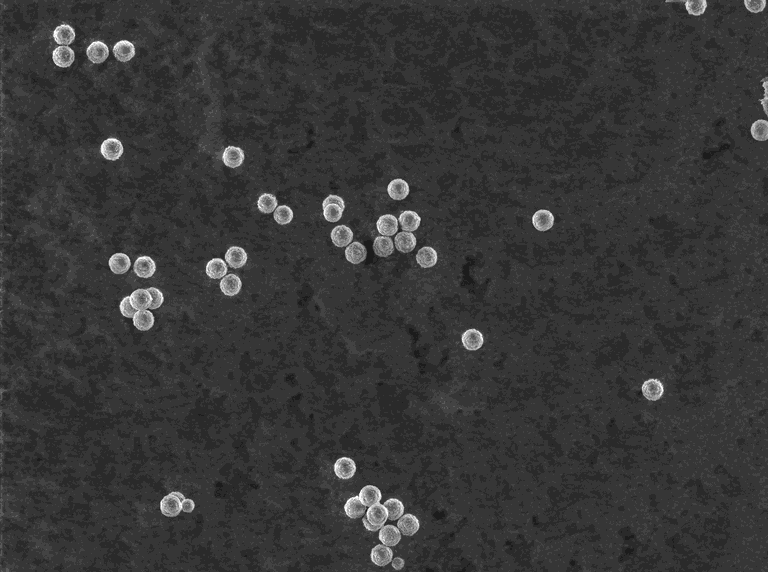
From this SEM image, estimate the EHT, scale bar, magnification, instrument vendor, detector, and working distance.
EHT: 5 kV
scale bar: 20000 nm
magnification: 1.7 K X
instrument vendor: Tescan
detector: SE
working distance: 10.32 mm